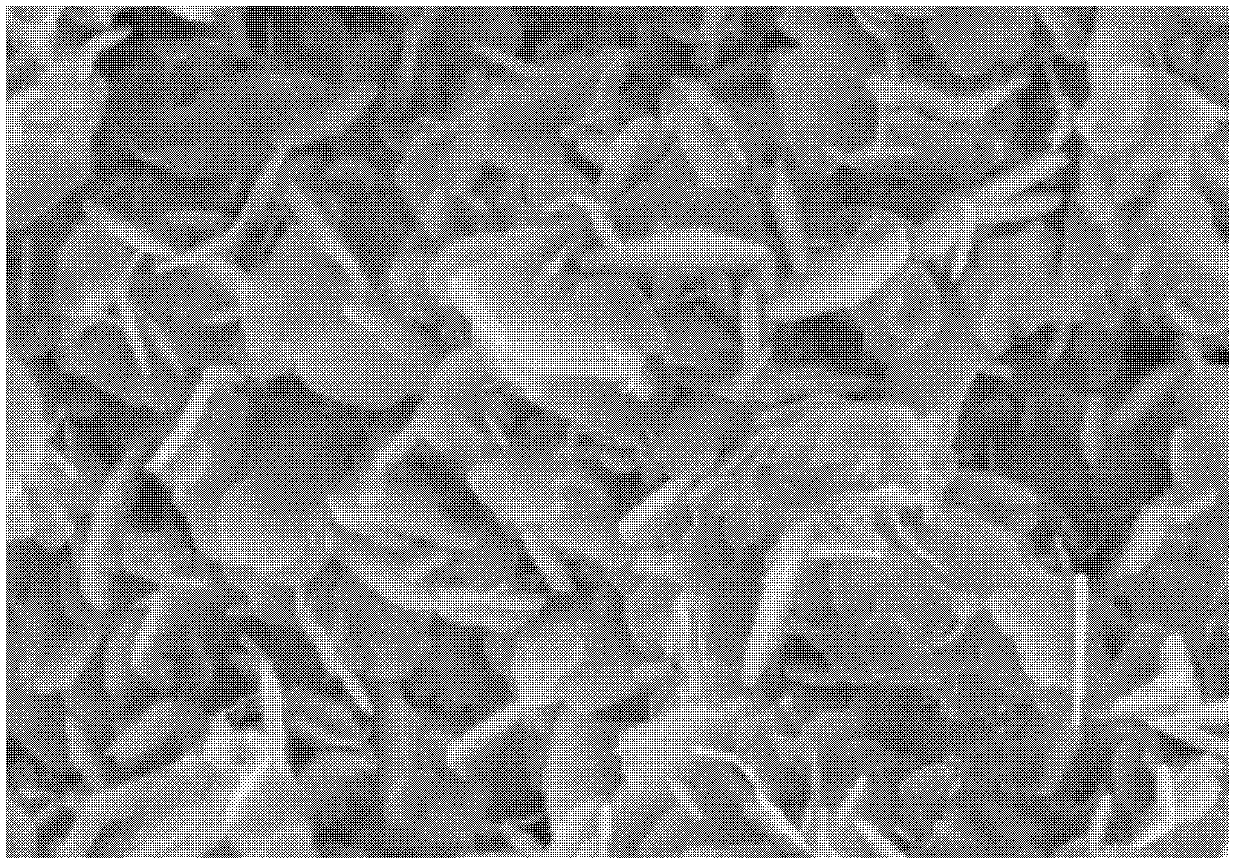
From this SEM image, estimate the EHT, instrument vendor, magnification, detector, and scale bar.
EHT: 4 kV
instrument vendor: Hitachi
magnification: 80 K X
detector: SE(U)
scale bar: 500 nm